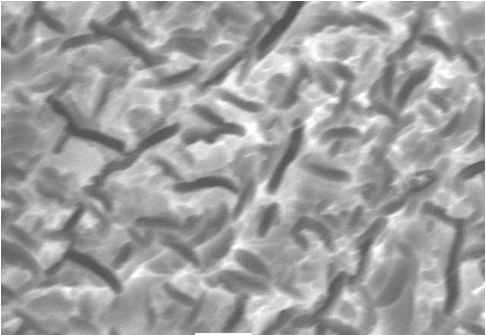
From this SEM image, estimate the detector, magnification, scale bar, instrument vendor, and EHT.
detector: SEI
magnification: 3 K X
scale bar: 5000 nm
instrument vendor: JEOL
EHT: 20 kV